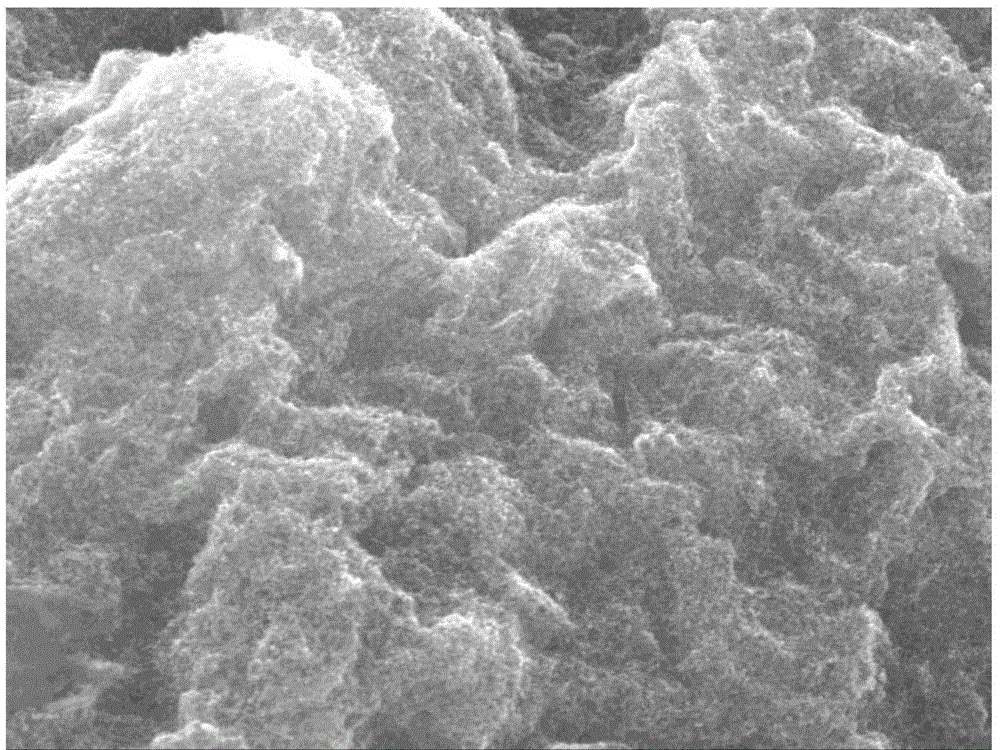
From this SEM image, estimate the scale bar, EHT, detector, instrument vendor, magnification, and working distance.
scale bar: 1000 nm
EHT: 15 kV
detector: SEI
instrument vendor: JEOL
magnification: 10 K X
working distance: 10.1 mm